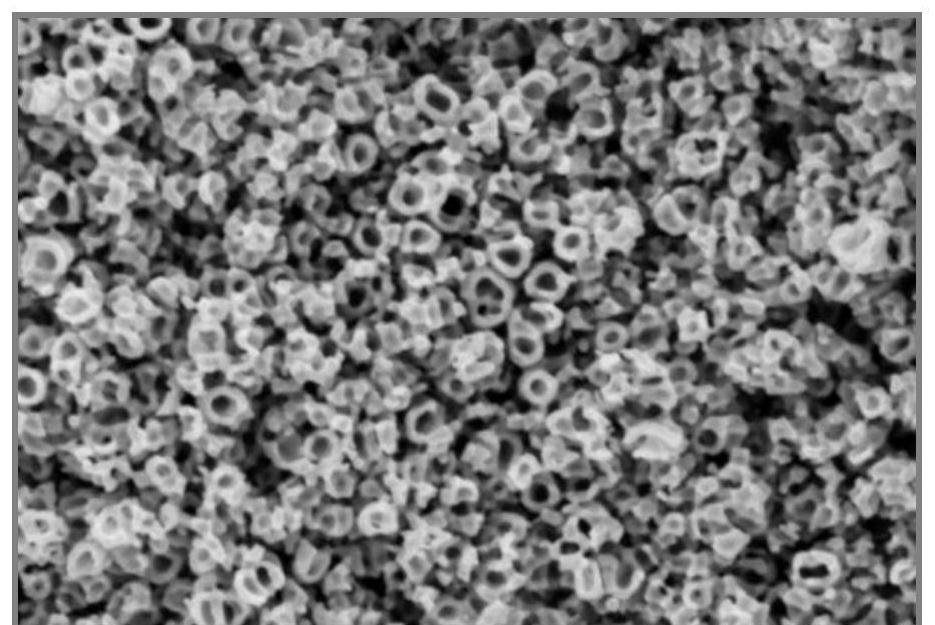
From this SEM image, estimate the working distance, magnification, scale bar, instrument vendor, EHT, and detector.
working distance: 9.1 mm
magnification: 50 K X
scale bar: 1000 nm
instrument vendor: Hitachi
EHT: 2 kV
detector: SE(UL)